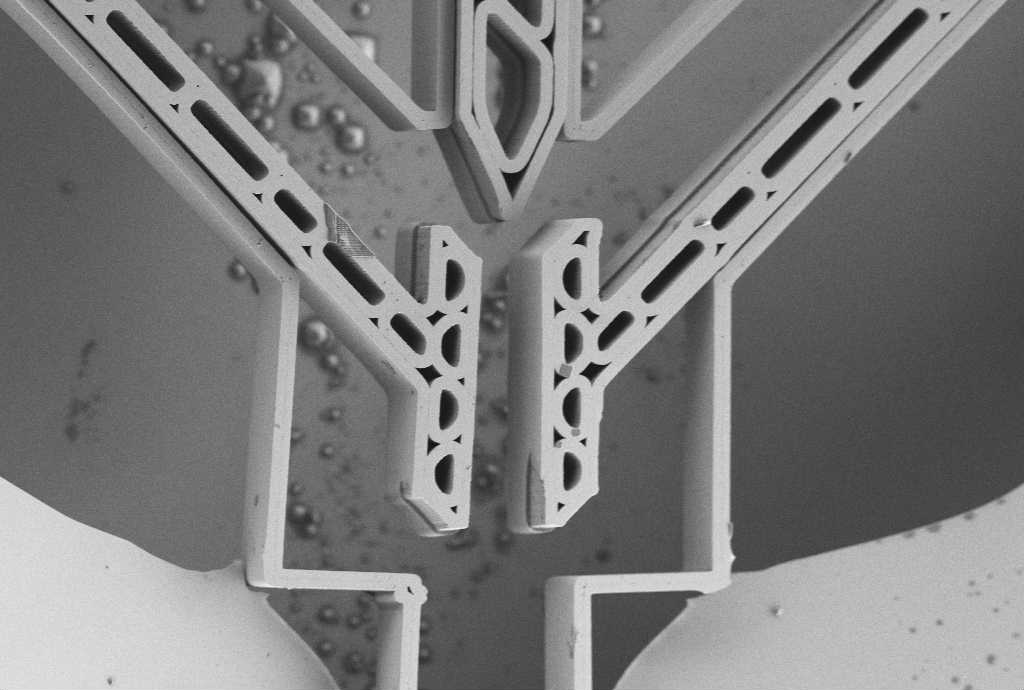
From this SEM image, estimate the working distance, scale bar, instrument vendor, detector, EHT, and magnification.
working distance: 6 mm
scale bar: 10000 nm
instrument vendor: Zeiss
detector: SE2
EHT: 1 kV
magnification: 0.572 K X